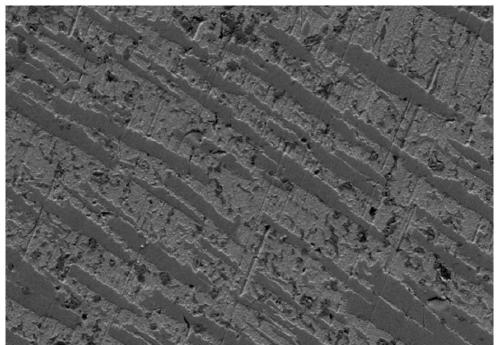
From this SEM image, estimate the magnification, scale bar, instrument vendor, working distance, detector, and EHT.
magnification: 0.5 K X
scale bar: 10000 nm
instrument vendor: Zeiss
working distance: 8 mm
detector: SE2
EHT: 10 kV